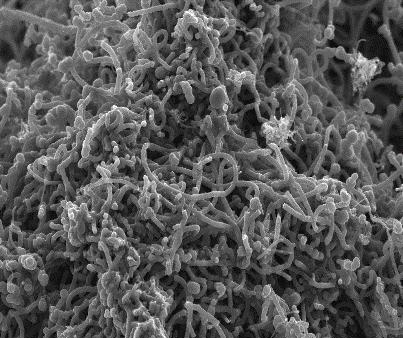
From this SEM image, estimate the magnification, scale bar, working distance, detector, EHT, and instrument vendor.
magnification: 25 K X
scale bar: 1000 nm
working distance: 3.2 mm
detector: InLens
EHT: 5 kV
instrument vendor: Zeiss